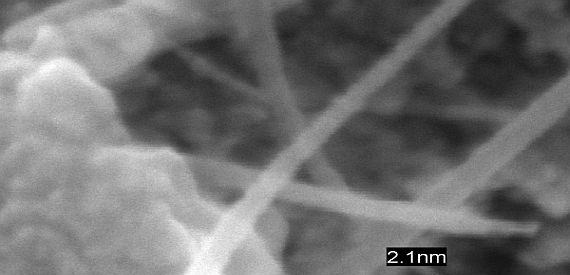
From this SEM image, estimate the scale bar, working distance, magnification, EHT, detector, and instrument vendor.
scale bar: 50 nm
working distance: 1.5 mm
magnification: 801 K X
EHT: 3 kV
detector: SE(U)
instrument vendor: Hitachi